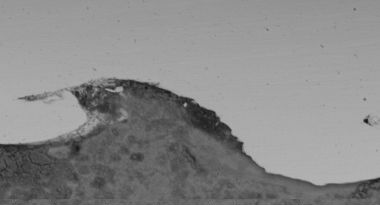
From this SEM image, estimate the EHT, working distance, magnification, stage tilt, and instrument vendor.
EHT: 10 kV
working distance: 13.6 mm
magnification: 2 K X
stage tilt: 0°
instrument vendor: FEI